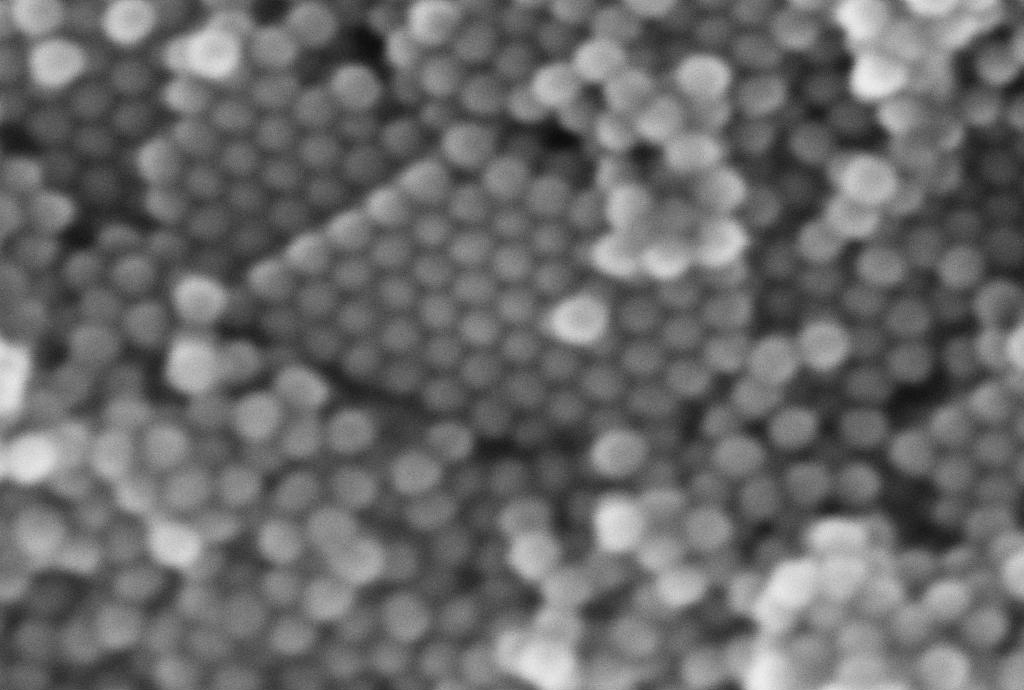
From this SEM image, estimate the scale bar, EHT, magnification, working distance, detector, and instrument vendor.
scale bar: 20 nm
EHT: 5 kV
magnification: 1000 K X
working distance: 2 mm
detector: InLens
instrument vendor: Zeiss